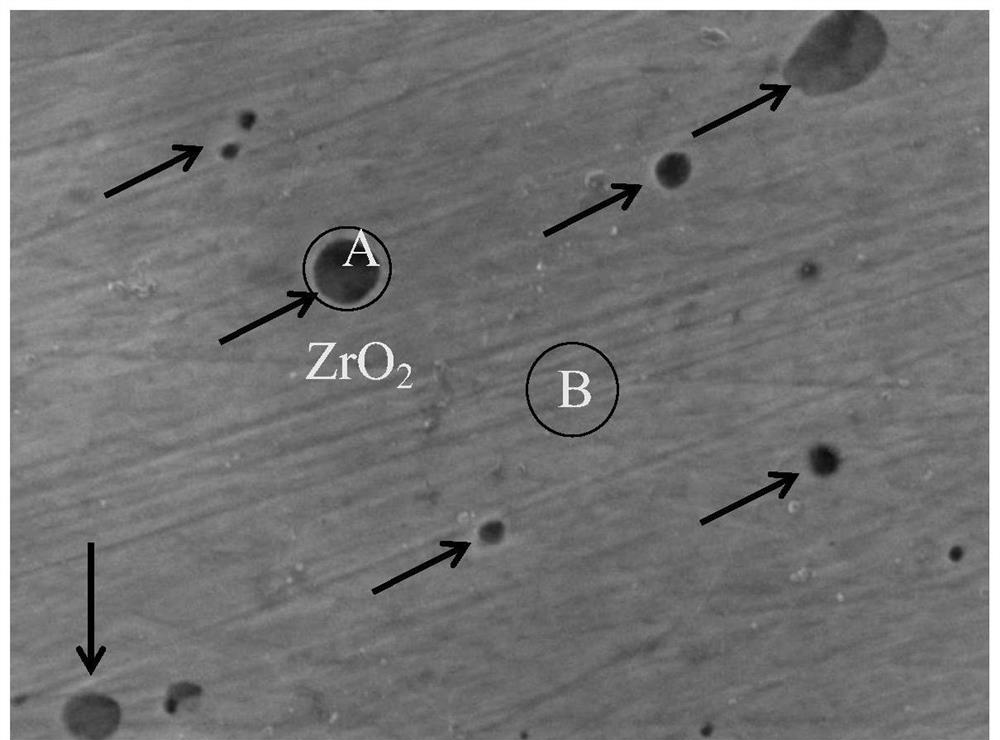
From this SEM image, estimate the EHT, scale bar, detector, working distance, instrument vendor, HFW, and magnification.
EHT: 10 kV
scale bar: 1000 nm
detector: SED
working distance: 10.6 mm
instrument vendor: JEOL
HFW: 11.6 µm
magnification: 11 K X